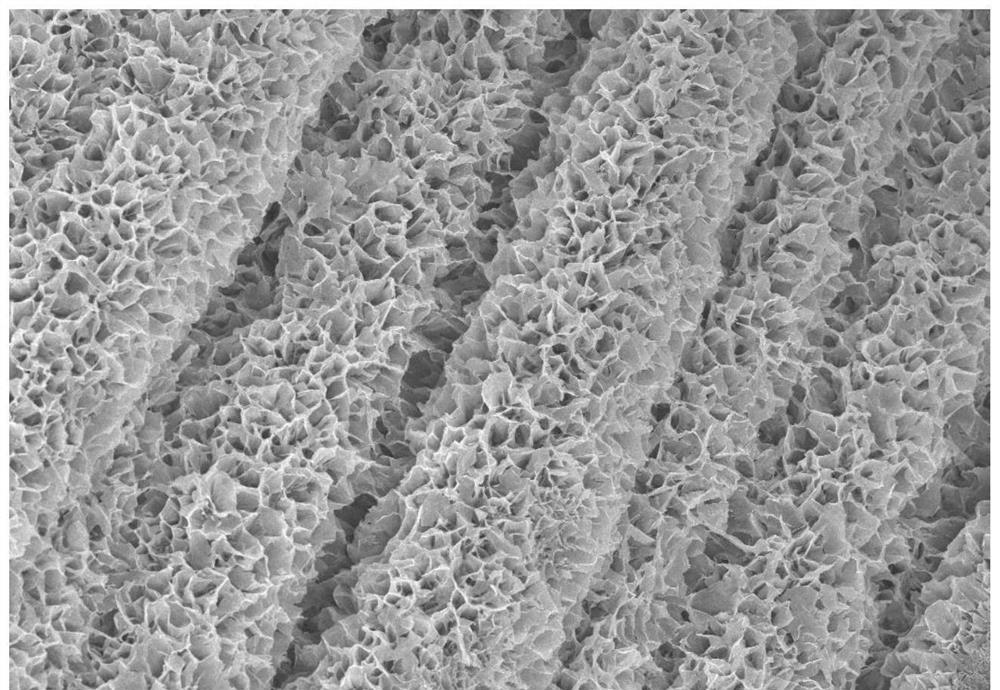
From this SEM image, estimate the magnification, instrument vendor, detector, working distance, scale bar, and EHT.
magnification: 2 K X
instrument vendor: Zeiss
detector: InLens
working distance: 8.3 mm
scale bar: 10000 nm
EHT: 5 kV